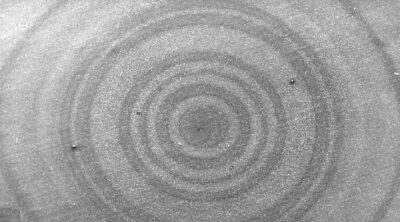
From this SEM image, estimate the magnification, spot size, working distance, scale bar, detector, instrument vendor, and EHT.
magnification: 0.049 K X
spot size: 5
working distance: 11.4 mm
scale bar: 1000000 nm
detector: SE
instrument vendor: FEI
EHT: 15 kV